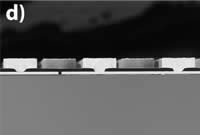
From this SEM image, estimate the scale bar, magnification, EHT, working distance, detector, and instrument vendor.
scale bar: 200000 nm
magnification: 0.25 K X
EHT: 10 kV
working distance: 5 mm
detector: BSE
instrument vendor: Hitachi